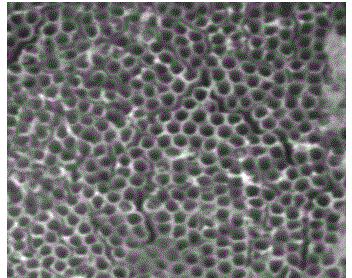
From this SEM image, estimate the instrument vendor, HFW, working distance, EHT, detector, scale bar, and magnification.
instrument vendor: Tescan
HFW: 2.77 µm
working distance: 5.55 mm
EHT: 30 kV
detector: SE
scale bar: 500 nm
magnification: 50 K X